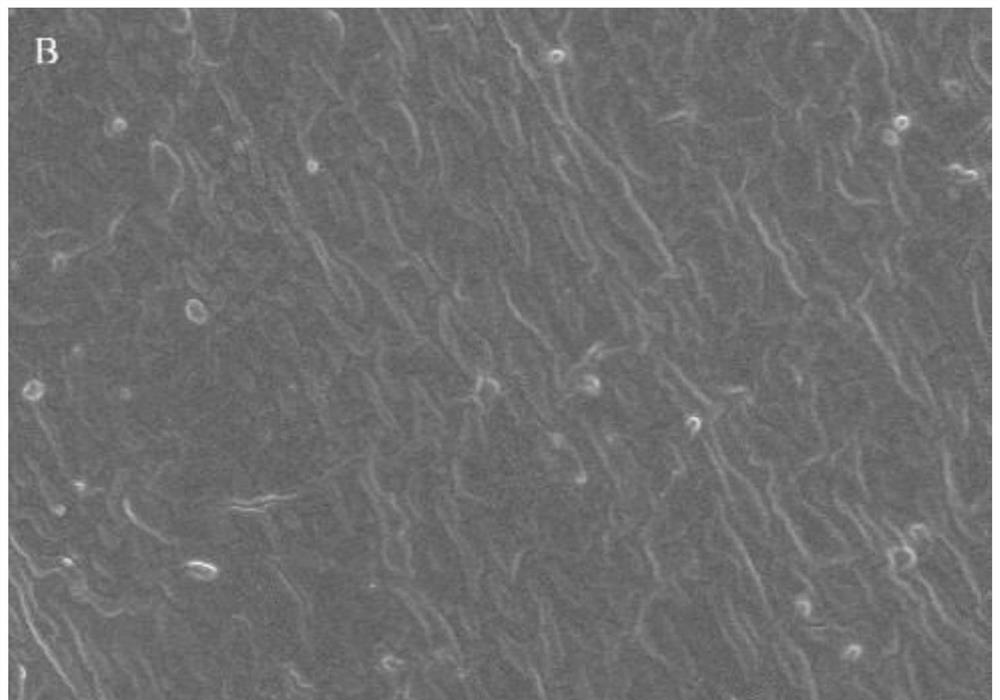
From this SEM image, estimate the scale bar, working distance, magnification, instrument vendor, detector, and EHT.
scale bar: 20000 nm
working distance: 9 mm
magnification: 2.2 K X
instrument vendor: Hitachi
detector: SE(U)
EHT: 5 kV